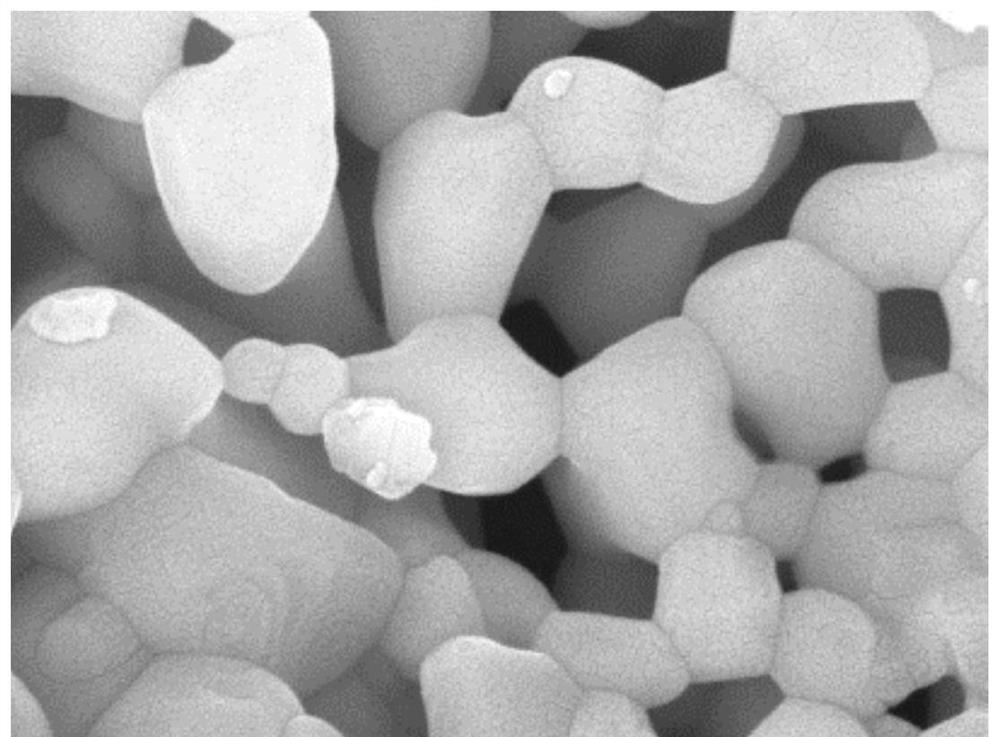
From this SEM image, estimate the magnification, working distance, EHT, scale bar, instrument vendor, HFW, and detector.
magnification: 110 K X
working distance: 15.56 mm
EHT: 15 kV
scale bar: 500 nm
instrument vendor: other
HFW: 2.54 µm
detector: SE